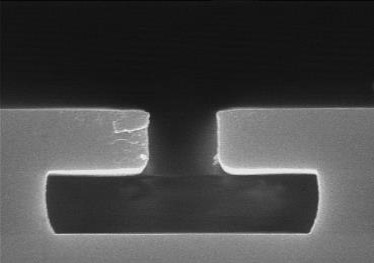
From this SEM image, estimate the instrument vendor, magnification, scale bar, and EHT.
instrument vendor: Hitachi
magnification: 25 K X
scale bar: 1200 nm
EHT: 15 kV